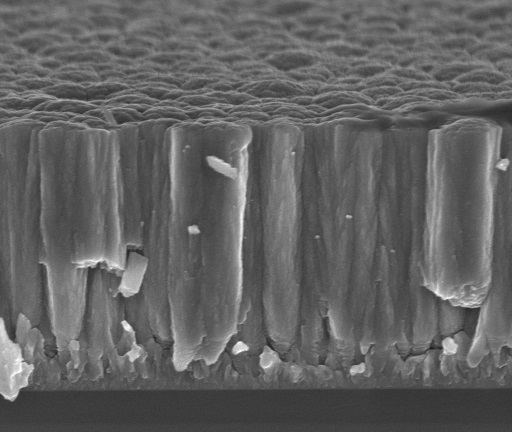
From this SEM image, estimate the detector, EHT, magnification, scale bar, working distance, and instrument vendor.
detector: TLD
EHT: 5 kV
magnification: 100 K X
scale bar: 500 nm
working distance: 4.8 mm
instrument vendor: FEI